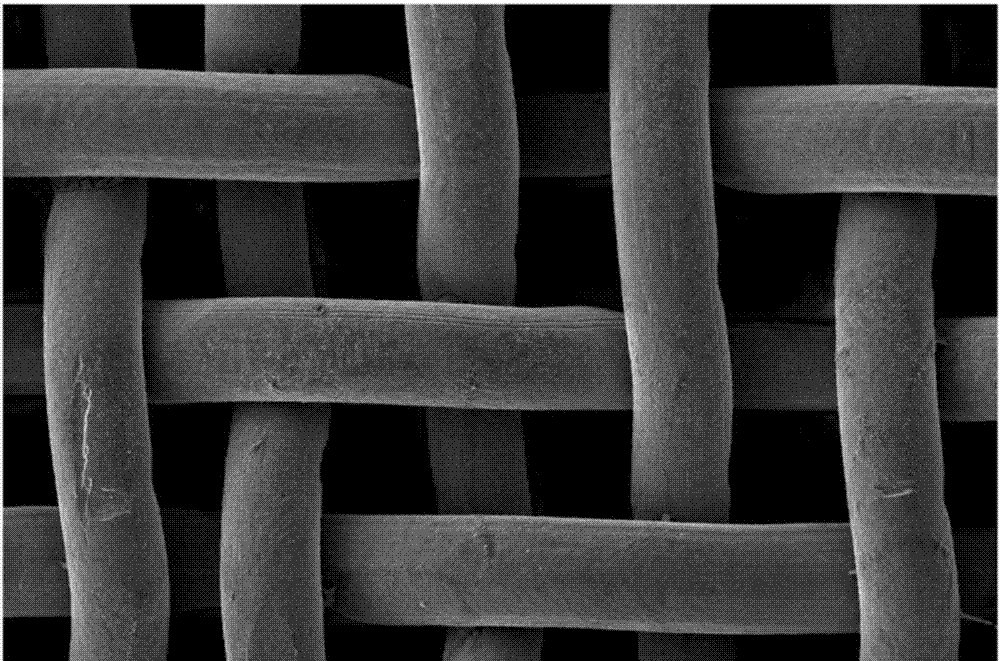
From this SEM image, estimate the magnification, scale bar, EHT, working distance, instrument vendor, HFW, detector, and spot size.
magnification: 0.5 K X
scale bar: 100000 nm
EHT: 10 kV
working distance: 10.3 mm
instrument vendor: FEI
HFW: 254 µm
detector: ETD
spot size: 3